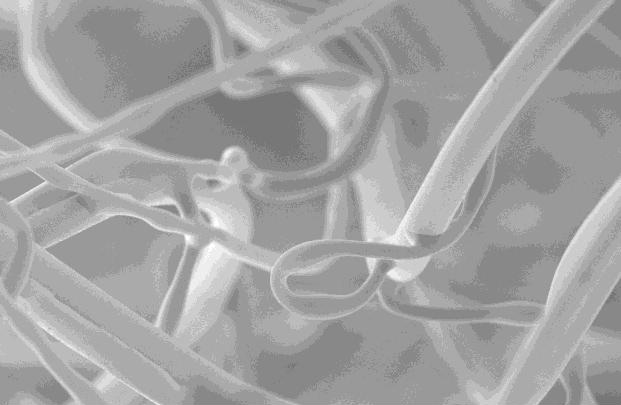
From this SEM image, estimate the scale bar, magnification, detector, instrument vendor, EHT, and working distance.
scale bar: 100000 nm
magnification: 0.5 K X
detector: SE2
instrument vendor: Zeiss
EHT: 5 kV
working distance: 9 mm